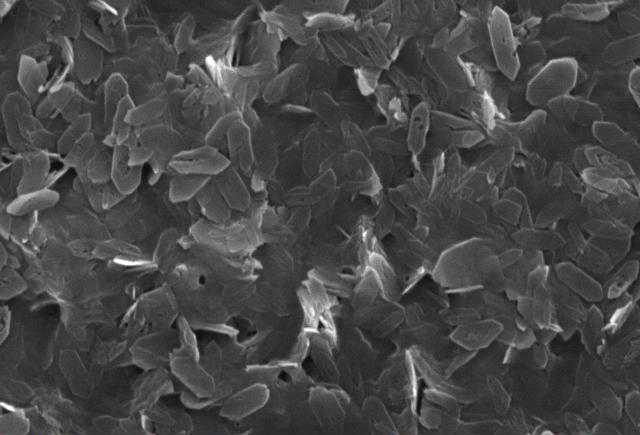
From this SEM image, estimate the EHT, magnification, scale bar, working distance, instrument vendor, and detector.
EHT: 20 kV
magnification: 5 K X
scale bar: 5000 nm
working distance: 23 mm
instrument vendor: JEOL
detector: SEI